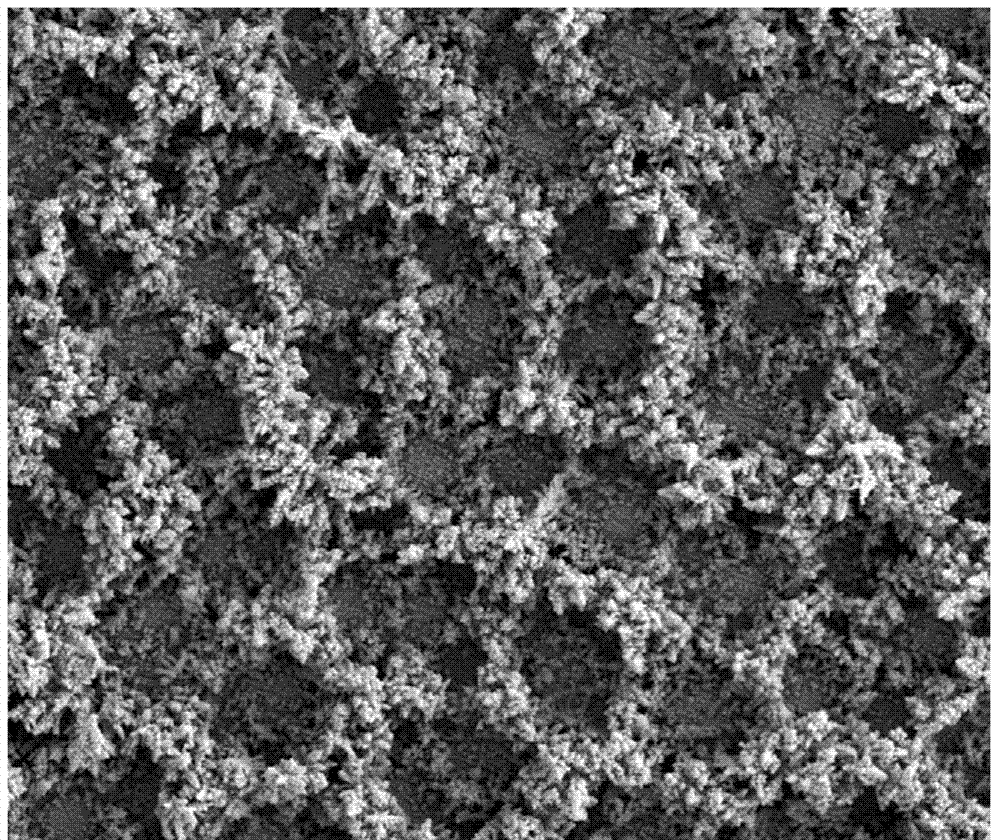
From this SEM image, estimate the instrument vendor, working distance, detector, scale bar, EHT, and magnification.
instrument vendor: FEI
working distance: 5.4 mm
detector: SE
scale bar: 100000 nm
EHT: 20 kV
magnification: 0.8 K X